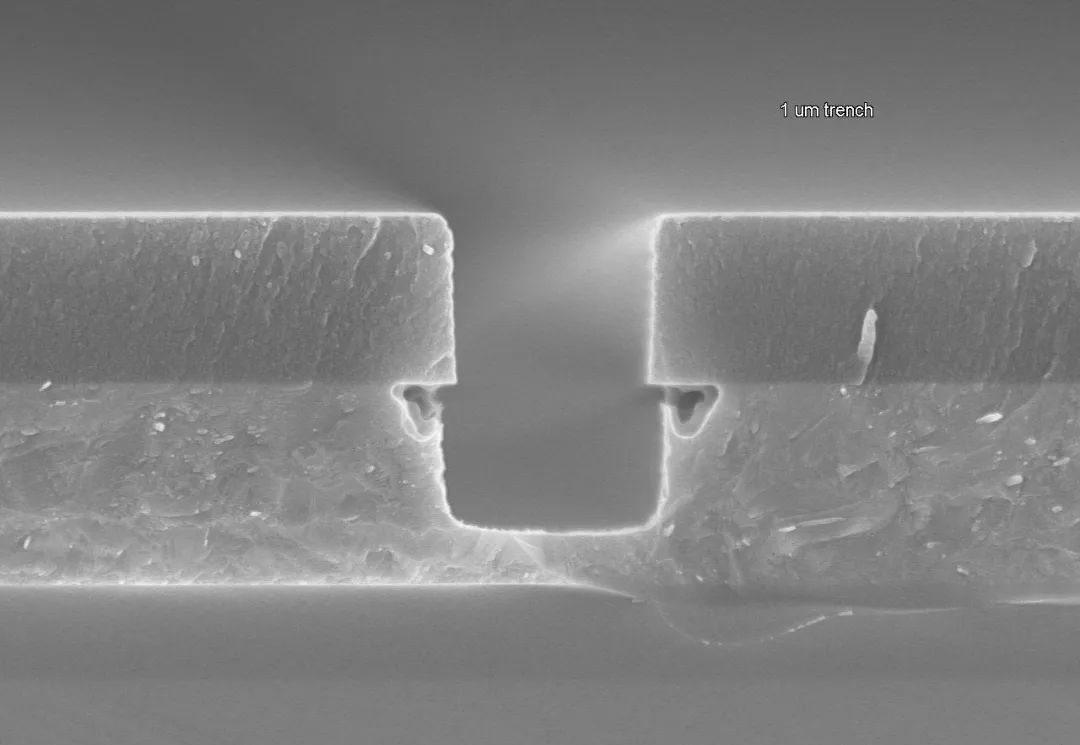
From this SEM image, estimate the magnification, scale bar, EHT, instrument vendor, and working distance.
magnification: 60 K X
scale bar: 2000 nm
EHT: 5 kV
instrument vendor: Hitachi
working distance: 8 mm